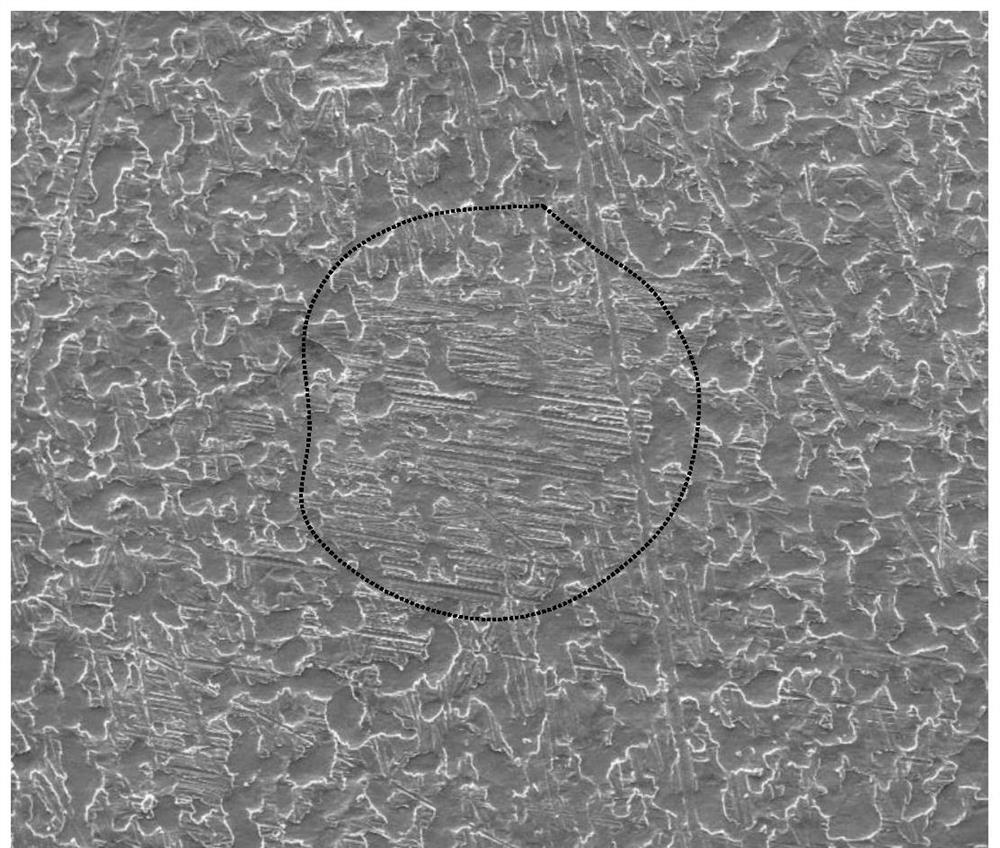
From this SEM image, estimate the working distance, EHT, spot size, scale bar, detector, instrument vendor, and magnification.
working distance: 12 mm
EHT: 20 kV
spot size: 5.5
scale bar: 500000 nm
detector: ETD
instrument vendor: FEI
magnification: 0.1 K X